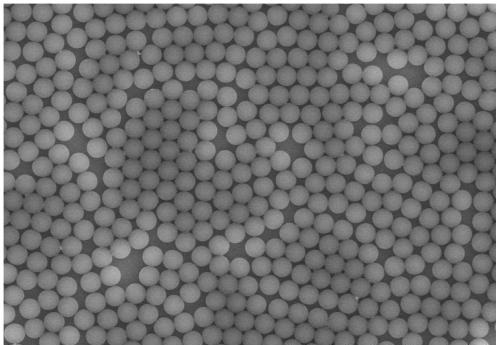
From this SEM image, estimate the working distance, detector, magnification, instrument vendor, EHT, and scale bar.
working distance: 13.5 mm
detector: SE(UL)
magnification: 5 K X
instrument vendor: Hitachi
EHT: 10 kV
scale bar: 10000 nm